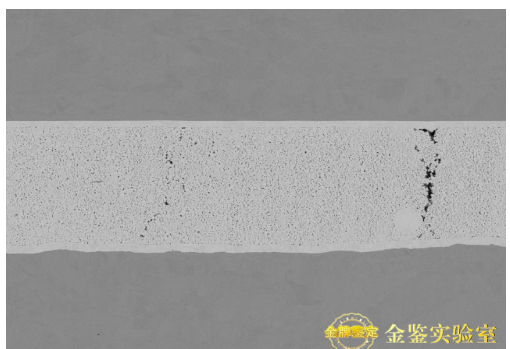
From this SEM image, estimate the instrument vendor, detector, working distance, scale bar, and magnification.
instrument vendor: Hitachi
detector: NL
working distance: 7.5 mm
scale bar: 100000 nm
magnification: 1 K X